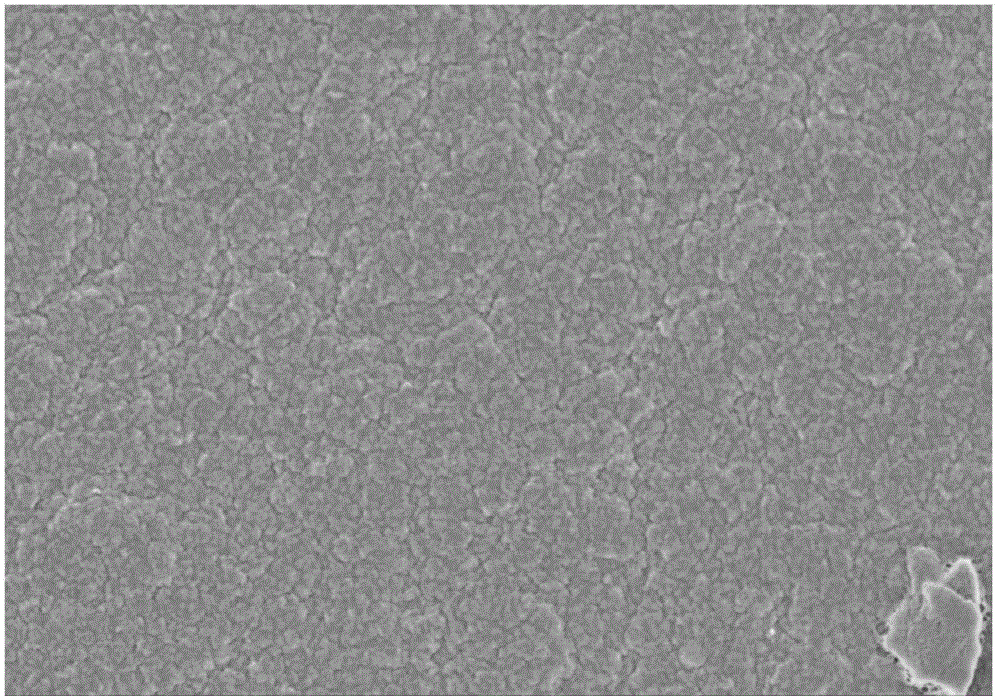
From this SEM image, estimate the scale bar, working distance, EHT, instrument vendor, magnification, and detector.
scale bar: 5000 nm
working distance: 5 mm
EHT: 2 kV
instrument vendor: Hitachi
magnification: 10 K X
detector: SE(M)